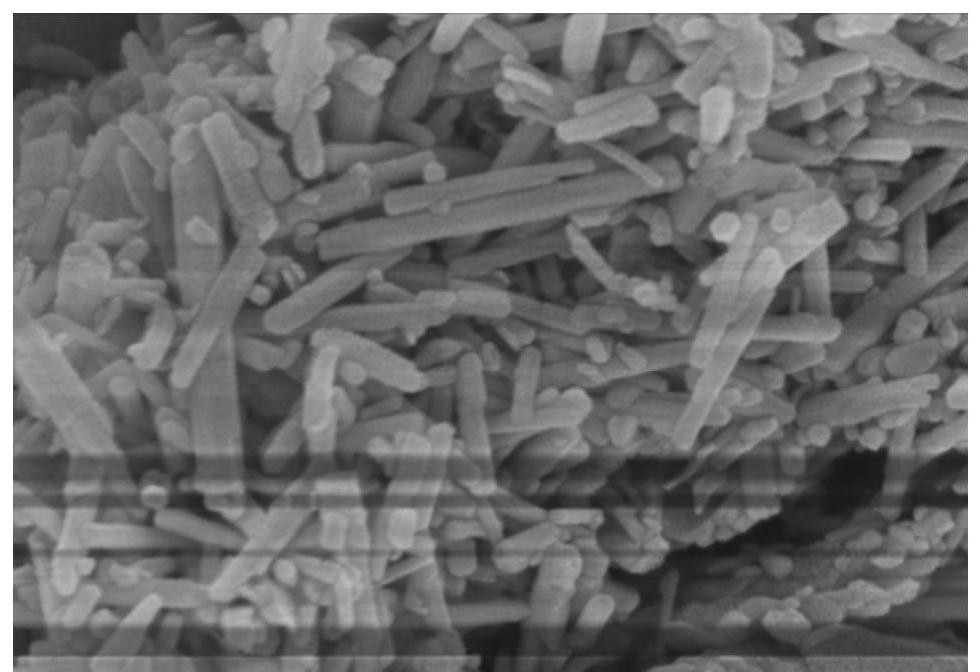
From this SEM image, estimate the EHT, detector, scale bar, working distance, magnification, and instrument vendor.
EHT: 5 kV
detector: SE(U)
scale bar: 500 nm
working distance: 4.9 mm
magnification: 100 K X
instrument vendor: Hitachi